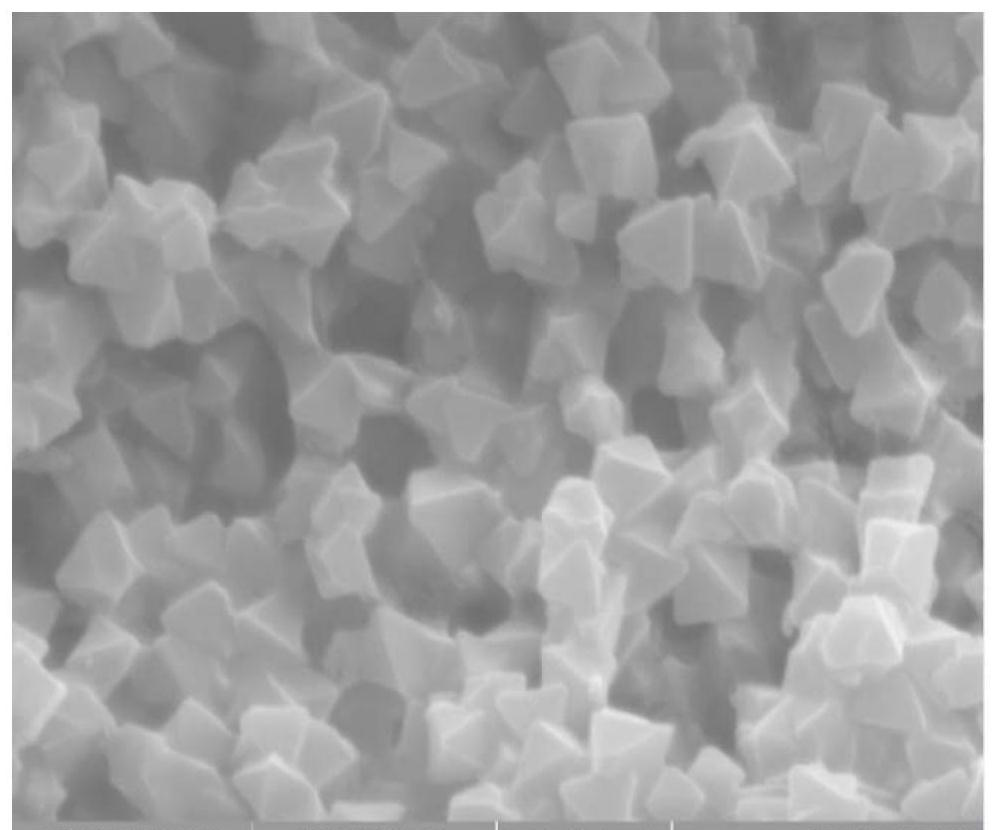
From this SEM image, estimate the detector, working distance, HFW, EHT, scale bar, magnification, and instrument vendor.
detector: SE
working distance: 5.94 mm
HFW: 5.54 µm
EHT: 10 kV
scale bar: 1000 nm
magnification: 50 K X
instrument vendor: Tescan